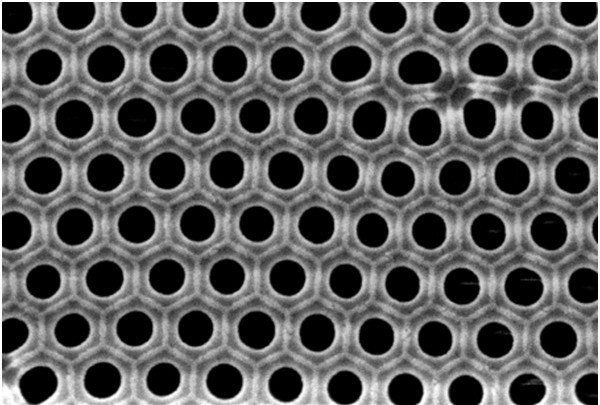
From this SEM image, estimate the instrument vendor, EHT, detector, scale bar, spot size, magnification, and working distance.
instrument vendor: JEOL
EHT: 20 kV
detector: SEI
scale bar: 500 nm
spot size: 15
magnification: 43 K X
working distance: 10 mm